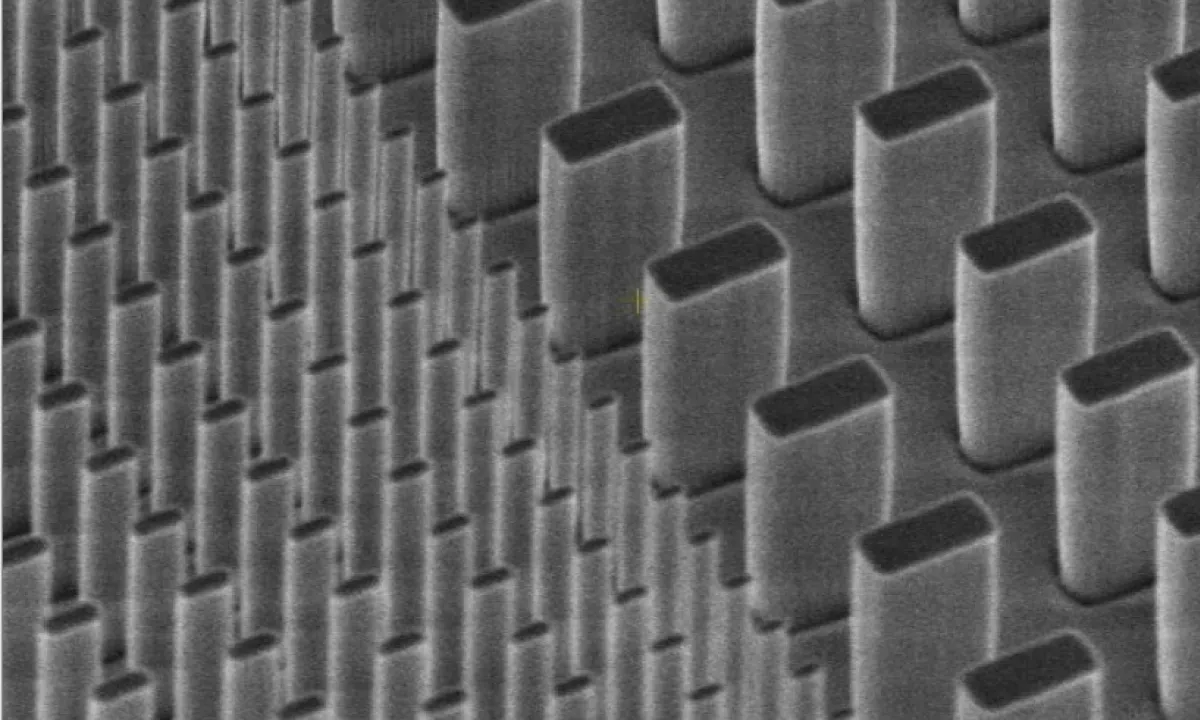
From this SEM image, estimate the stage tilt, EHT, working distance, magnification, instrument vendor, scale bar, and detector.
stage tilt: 45°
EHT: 2 kV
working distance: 4.8 mm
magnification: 40 K X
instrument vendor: FEI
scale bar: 1000 nm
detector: TLD